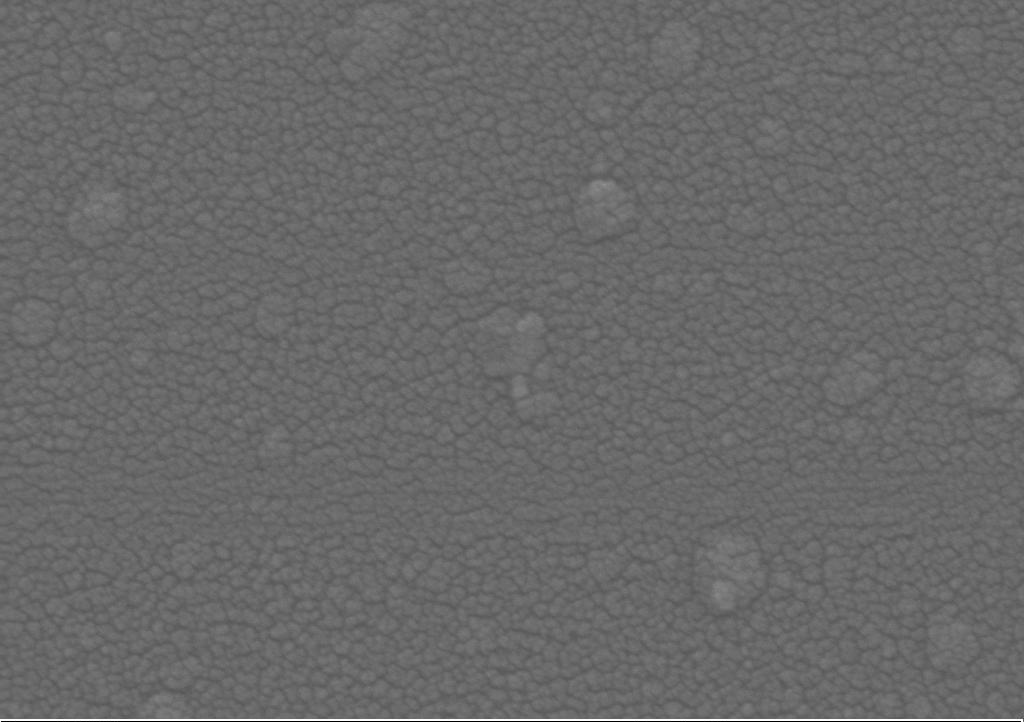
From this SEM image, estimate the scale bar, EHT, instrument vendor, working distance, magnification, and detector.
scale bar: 200 nm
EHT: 15 kV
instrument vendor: Zeiss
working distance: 7.4 mm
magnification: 150 K X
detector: SE2